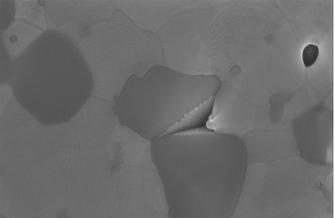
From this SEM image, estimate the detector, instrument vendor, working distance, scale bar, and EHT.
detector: InLens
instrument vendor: Zeiss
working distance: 3 mm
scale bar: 100 nm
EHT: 10 kV